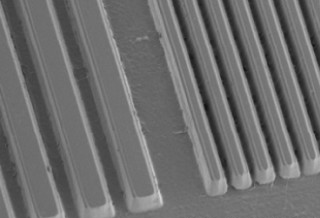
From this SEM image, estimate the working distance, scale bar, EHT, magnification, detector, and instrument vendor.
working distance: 7.7 mm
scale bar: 50000 nm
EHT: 2 kV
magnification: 1 K X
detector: SE(M)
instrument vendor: Hitachi